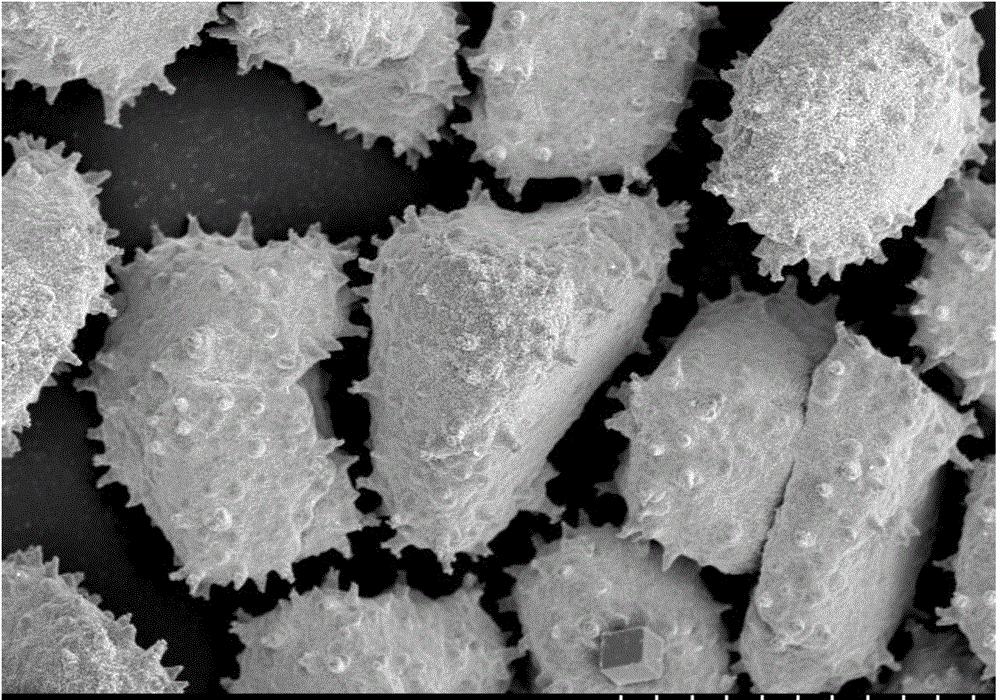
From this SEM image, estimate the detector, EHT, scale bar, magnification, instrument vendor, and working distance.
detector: SE(M)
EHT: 5 kV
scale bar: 30000 nm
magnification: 1.5 K X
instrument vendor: Hitachi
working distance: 8.8 mm